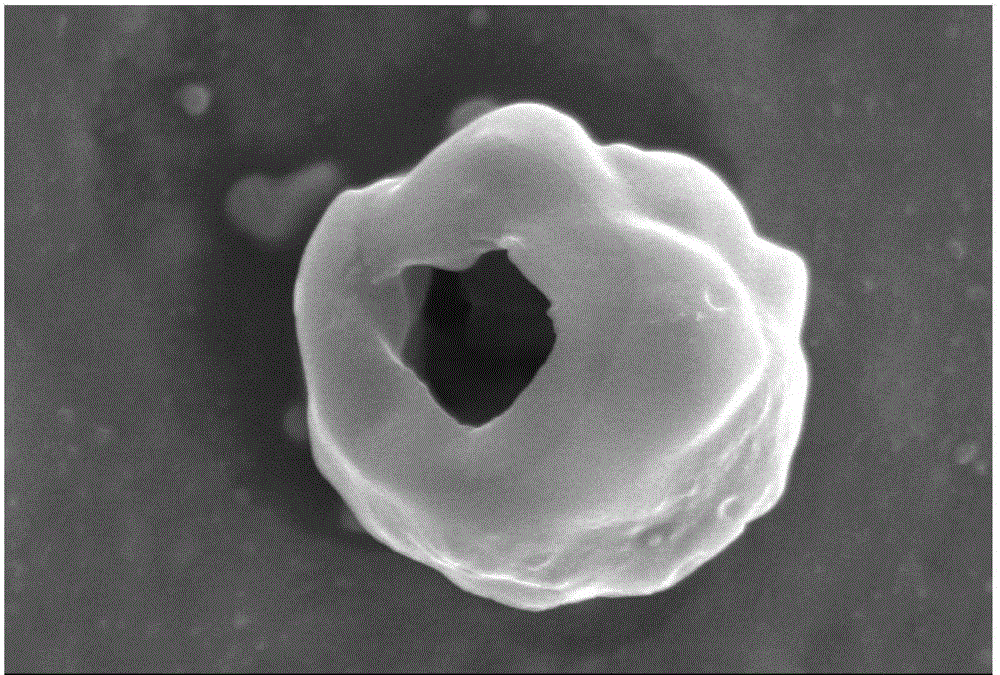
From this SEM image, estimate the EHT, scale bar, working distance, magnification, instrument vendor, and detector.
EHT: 10 kV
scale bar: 5000 nm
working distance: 13.6 mm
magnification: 8 K X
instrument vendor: Hitachi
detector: SE